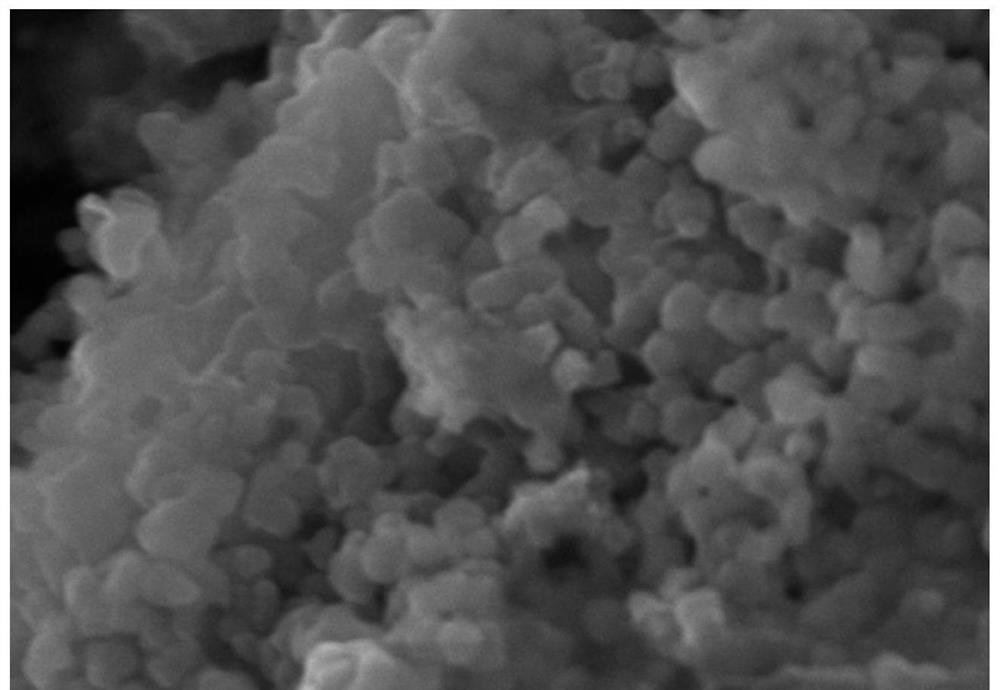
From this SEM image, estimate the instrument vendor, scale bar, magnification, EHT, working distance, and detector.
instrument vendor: Hitachi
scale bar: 500 nm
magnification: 100 K X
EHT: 10 kV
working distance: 4.8 mm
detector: SE(M)